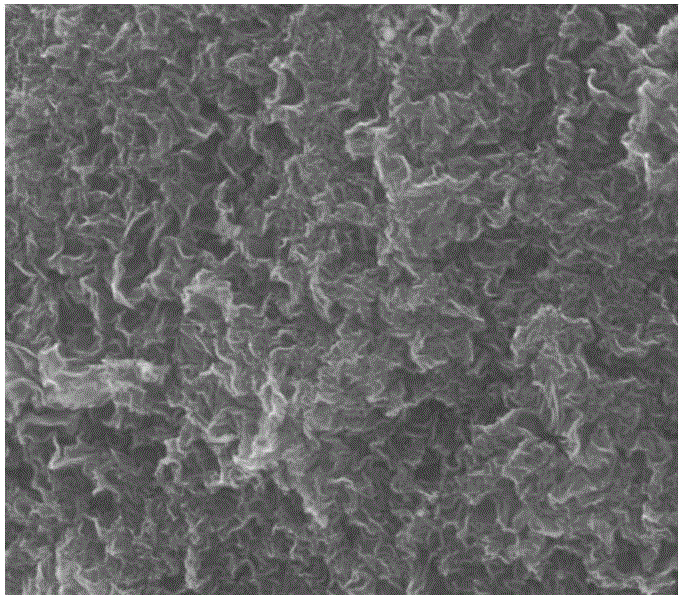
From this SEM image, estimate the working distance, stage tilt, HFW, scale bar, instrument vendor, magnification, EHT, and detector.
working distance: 9.9 mm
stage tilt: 0°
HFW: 18.6 µm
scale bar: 5000 nm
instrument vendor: FEI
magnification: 16 K X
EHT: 5 kV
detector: ETD